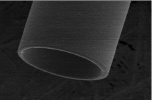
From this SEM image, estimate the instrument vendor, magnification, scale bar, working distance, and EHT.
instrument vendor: JEOL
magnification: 0.2 K X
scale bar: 100000 nm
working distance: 15 mm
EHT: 5 kV